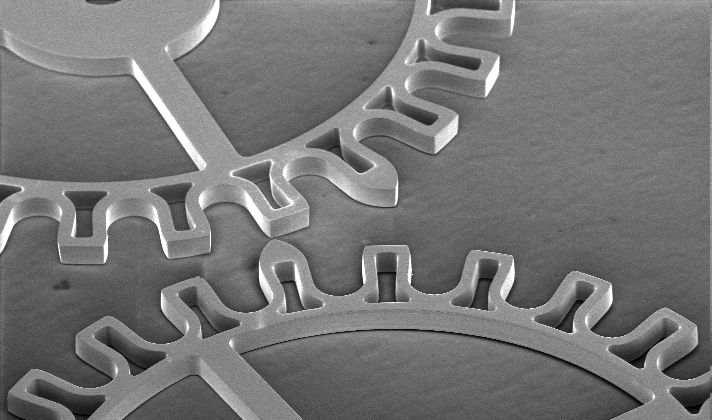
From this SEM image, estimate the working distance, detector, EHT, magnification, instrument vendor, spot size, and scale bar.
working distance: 29.4 mm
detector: SE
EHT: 15 kV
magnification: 0.033 K X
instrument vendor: FEI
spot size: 6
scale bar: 1000000 nm